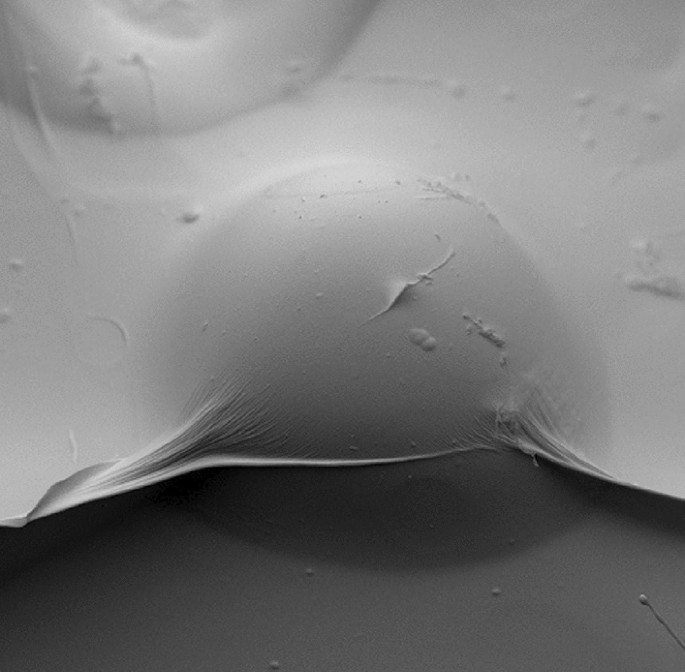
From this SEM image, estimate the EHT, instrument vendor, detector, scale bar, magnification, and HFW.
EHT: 10 kV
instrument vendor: Thermo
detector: BSD Topo B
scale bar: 50000 nm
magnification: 1.45 K X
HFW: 185 µm